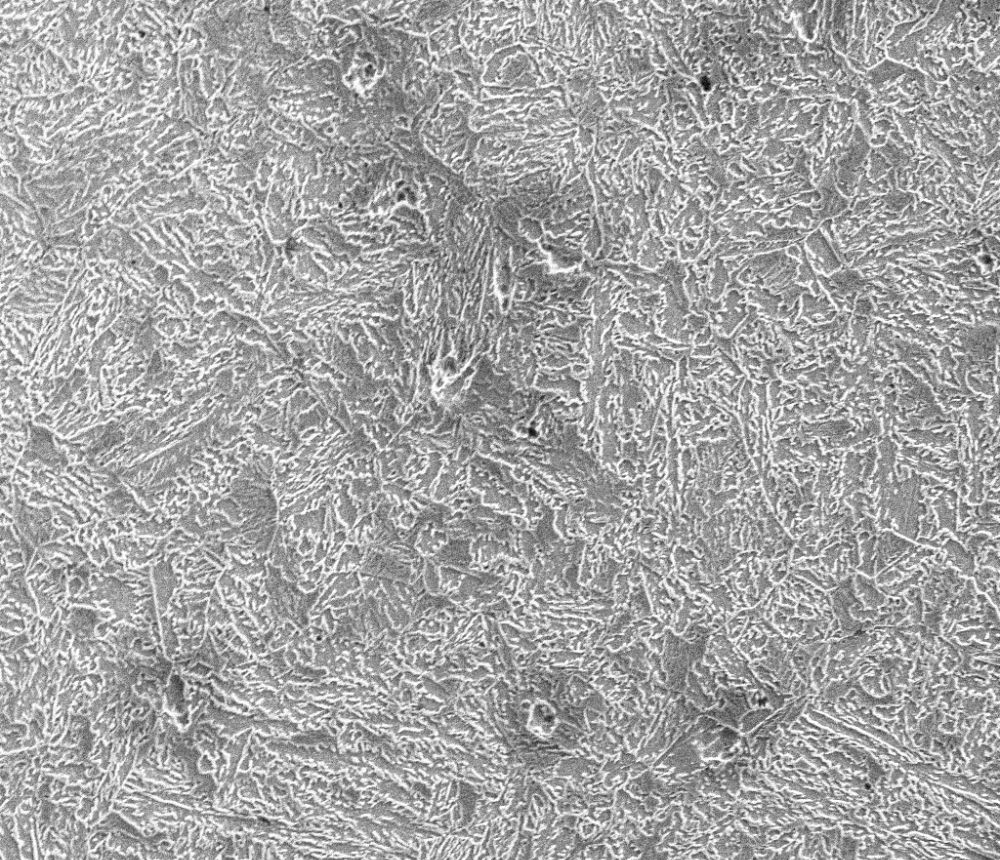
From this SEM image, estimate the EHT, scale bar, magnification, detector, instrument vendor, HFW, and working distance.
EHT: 10 kV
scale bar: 30000 nm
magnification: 2.5 K X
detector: ETD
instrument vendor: FEI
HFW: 120 µm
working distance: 5.4 mm